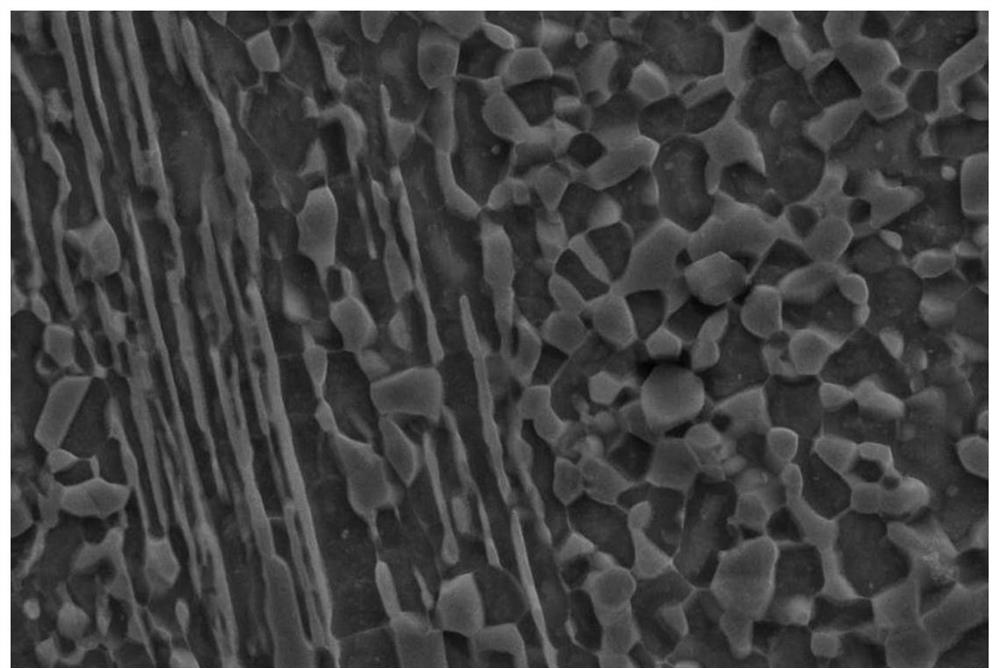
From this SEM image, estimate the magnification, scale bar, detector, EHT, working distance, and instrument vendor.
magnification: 20 K X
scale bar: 2000 nm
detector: SE2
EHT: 15 kV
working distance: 7.5 mm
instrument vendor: Zeiss